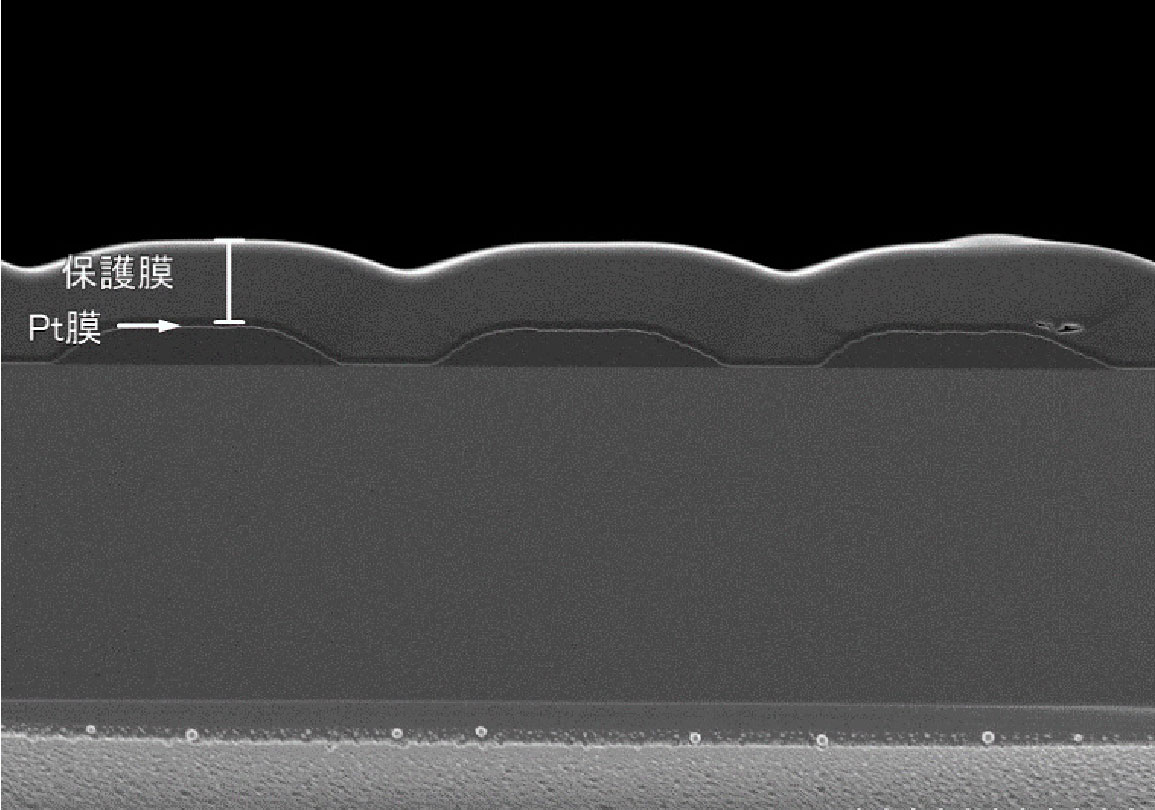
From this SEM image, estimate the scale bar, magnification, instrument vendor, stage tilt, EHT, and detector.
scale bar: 5000 nm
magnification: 6 K X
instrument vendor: Hitachi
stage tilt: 90°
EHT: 2 kV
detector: SE